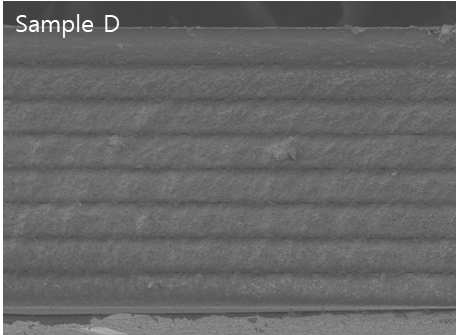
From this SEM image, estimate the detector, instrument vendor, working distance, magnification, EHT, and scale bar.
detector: SEI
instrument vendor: JEOL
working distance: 11.5 mm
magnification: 0.16 K X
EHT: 15 kV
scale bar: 100000 nm